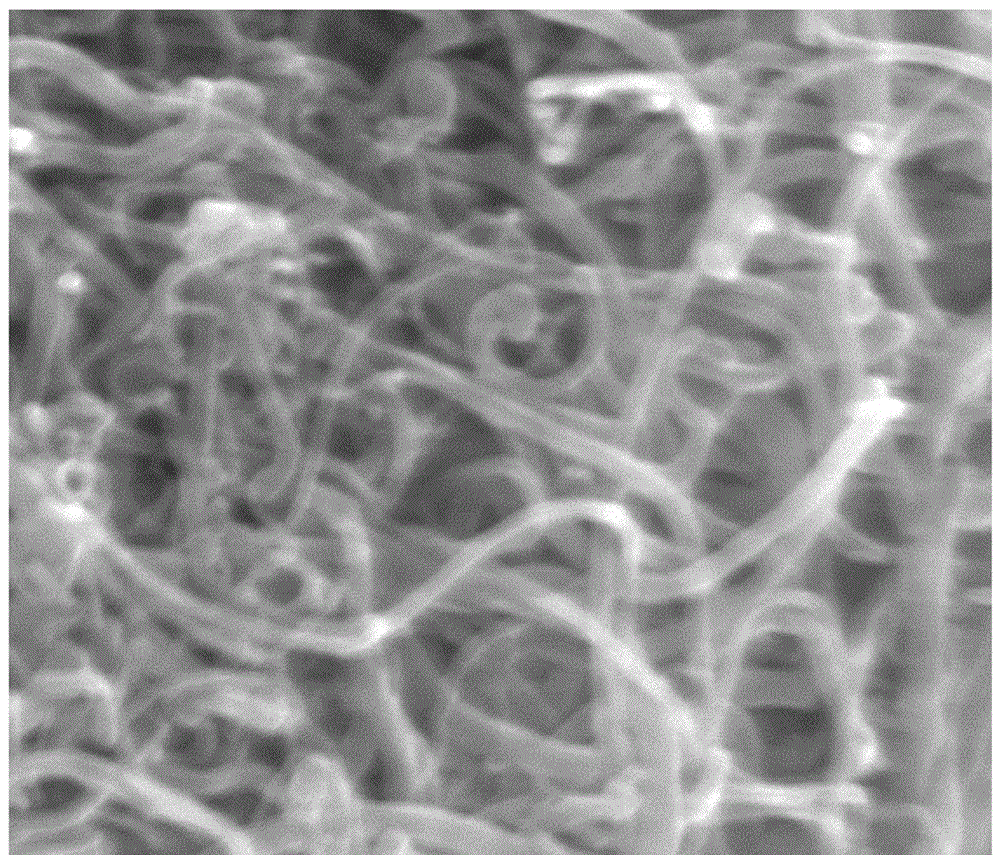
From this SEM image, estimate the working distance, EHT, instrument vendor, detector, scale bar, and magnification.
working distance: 5.8 mm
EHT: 10 kV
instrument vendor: FEI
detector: TLD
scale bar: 500 nm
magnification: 150 K X